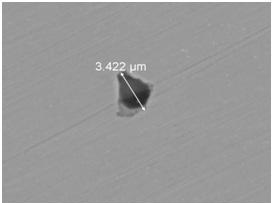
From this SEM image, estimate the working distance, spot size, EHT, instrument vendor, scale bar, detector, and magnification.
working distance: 11.9 mm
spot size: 4.5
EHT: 15 kV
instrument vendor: FEI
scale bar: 10000 nm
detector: BSED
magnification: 15 K X